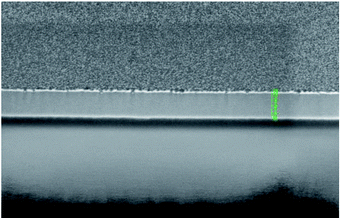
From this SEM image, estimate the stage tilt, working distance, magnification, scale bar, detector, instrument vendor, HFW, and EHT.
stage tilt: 52°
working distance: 4 mm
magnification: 200 K X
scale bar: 500 nm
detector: TLD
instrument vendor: FEI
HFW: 2.07 µm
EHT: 2 kV